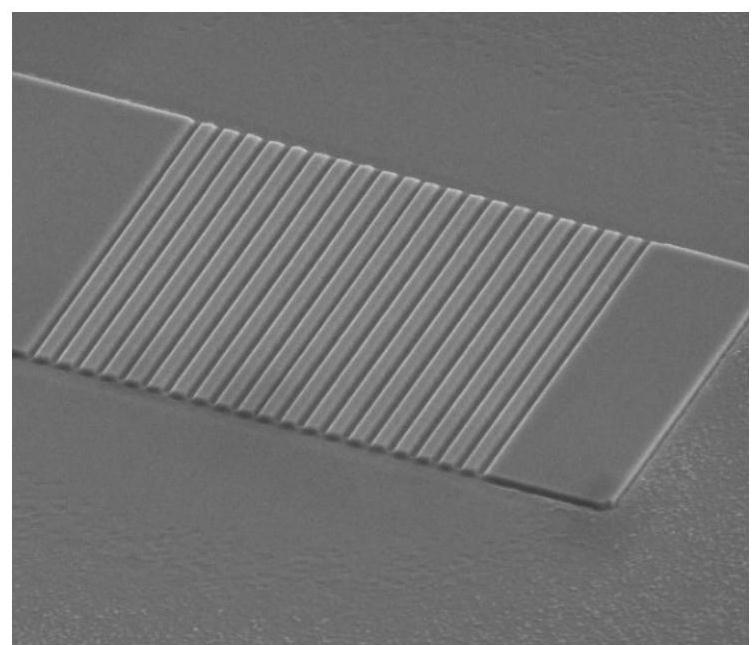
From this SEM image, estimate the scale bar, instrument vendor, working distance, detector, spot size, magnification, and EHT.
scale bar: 10000 nm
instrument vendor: FEI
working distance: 10.7 mm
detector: ETD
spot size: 4.5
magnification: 10 K X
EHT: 10 kV